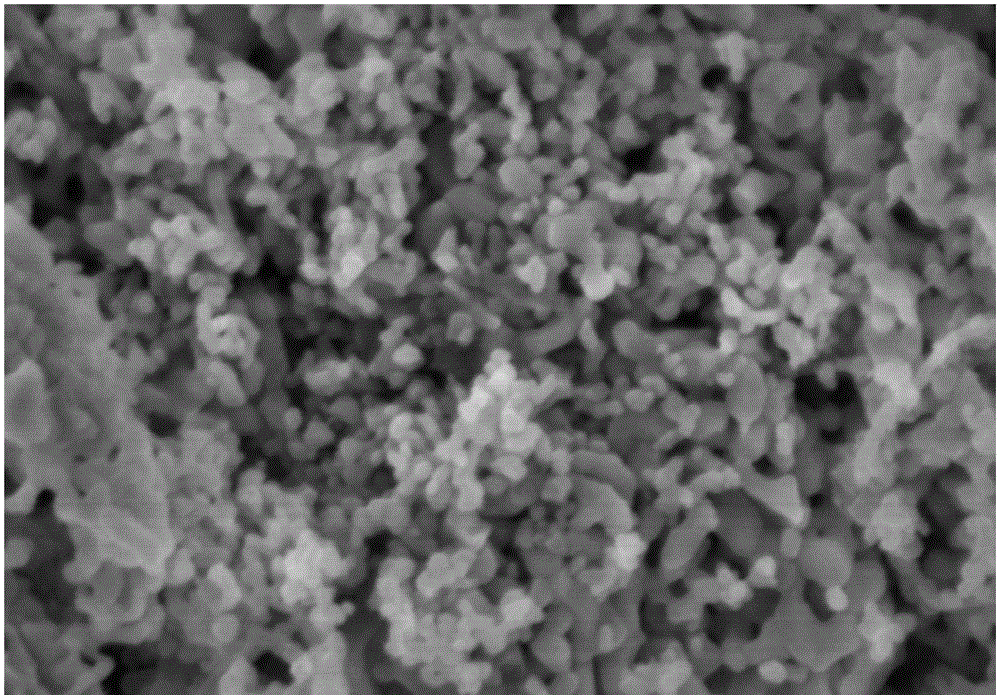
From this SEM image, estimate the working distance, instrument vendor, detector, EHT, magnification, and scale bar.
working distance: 8.2 mm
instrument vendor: Hitachi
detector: SE(U)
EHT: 3 kV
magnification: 110 K X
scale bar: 500 nm